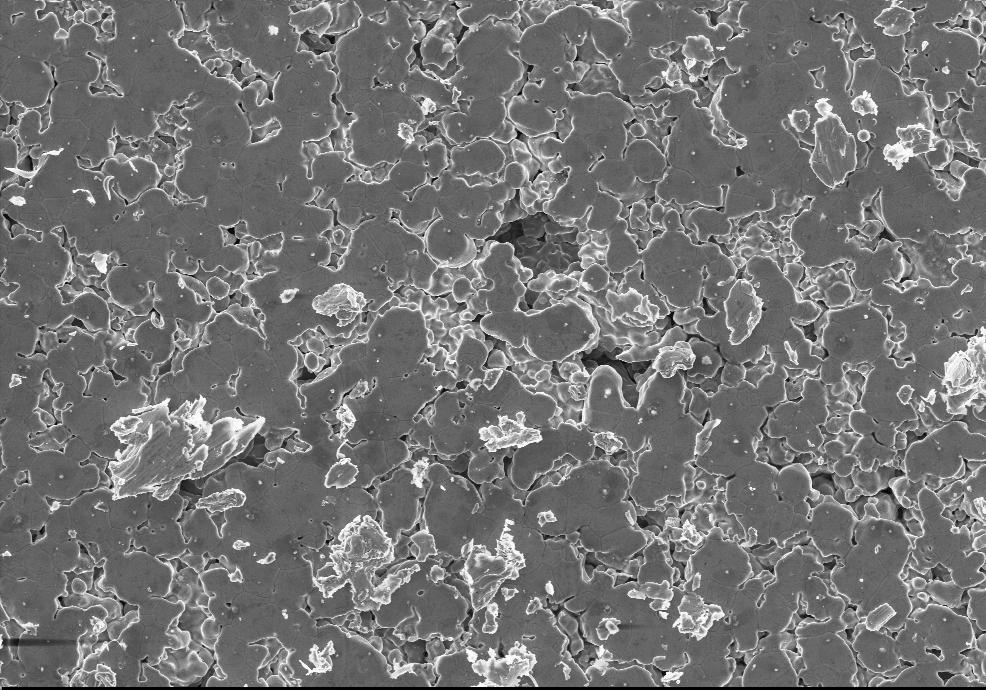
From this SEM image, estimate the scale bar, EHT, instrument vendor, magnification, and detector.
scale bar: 100000 nm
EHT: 20 kV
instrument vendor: Zeiss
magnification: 0.2 K X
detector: SE1